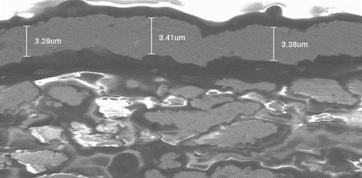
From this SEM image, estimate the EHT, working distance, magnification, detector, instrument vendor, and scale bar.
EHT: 5 kV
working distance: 8.8 mm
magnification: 10 K X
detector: SE(U)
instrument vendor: Hitachi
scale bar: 5000 nm